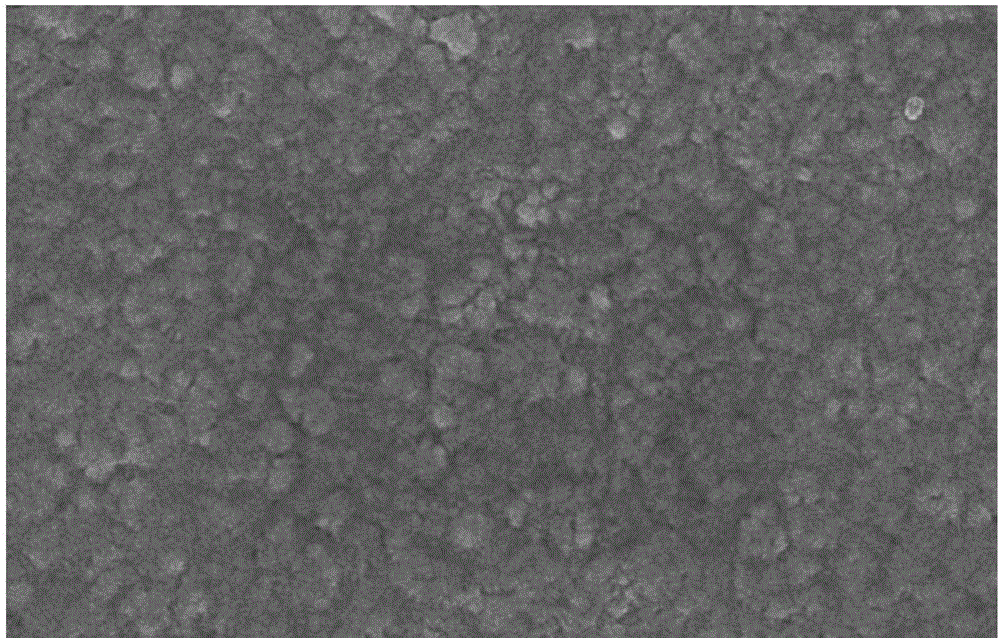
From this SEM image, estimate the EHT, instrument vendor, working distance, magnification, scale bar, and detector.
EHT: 5 kV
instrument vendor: Zeiss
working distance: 6.1 mm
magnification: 50 K X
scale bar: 200 nm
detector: InLens